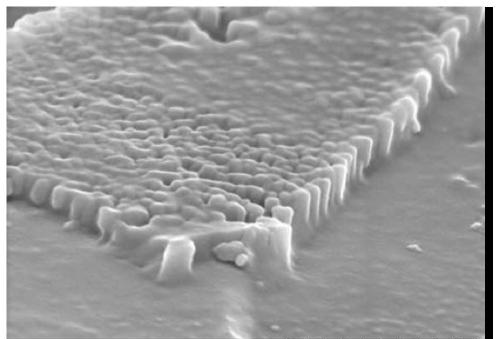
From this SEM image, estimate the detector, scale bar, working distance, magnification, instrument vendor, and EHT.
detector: SE(UL)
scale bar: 1000 nm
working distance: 8 mm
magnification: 50 K X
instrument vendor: Hitachi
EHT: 5 kV